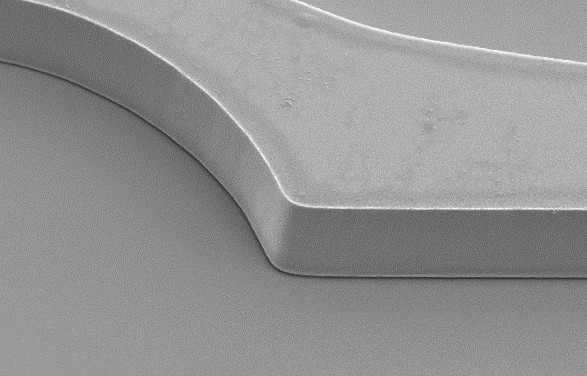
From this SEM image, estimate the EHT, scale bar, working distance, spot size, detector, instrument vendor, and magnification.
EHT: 5 kV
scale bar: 10000 nm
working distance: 7.6 mm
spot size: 3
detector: SE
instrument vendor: FEI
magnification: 2.203 K X